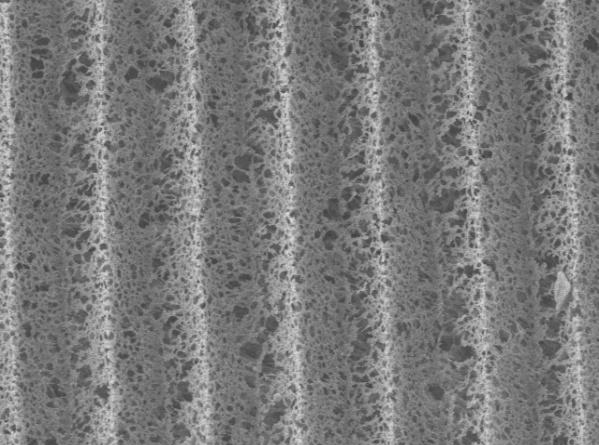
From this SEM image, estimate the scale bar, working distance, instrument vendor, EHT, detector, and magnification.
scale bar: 10000 nm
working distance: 8 mm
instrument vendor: JEOL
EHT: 10 kV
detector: LEI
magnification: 0.8 K X